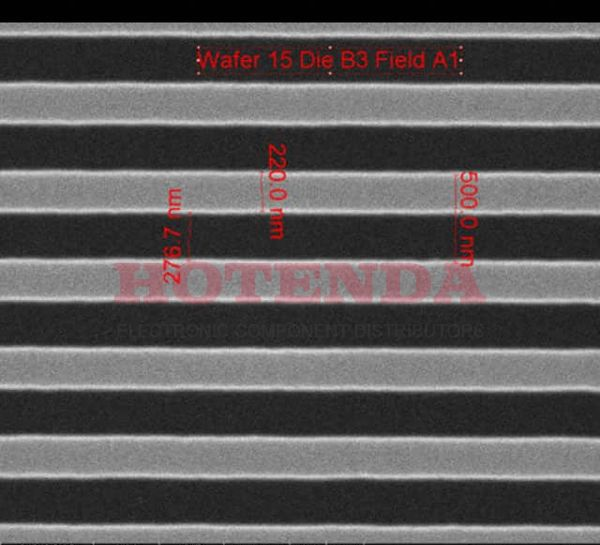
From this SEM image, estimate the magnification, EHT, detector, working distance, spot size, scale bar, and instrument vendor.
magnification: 75 K X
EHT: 10 kV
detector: ETD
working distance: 10.3 mm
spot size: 1.5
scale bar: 1000 nm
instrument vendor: FEI